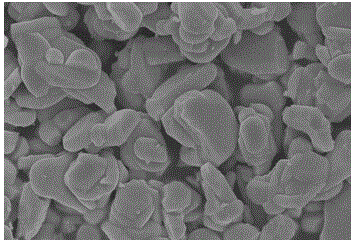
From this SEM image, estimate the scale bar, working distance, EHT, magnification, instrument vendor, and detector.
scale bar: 10000 nm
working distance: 8.3 mm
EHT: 15 kV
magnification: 4.5 K X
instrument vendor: Hitachi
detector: SE(U)